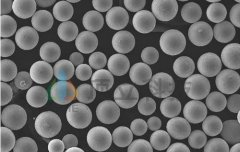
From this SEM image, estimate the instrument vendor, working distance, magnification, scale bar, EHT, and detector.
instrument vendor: Tescan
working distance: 5.46 mm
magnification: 0.5 K X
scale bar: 100000 nm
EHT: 30 kV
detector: SE